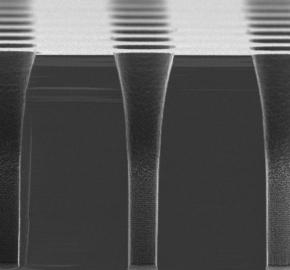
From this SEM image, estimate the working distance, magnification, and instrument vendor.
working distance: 15.2 mm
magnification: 1.3 K X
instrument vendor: JEOL